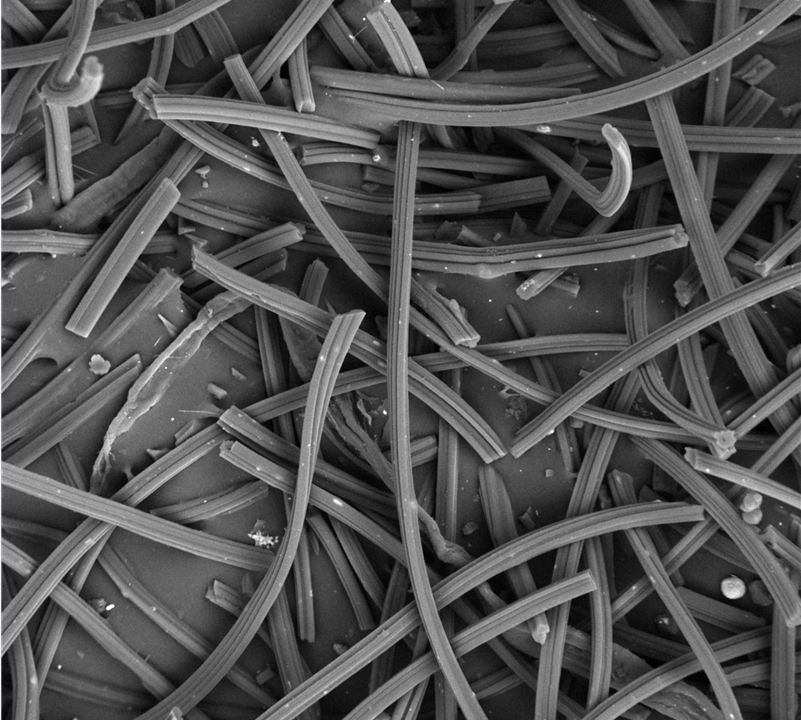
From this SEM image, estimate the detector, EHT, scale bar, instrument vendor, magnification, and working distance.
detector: BSE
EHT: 15 kV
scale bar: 50000 nm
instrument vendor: other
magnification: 1 K X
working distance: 8.6 mm